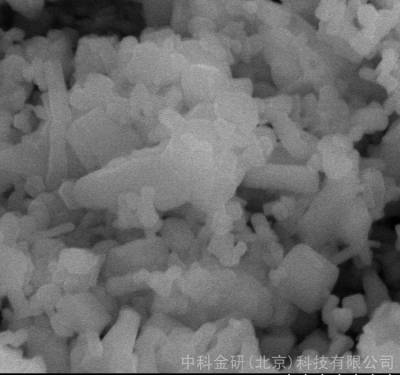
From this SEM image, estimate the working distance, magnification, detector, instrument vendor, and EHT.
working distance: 14 mm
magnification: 20 K X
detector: SE(M)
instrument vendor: Hitachi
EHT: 20 kV